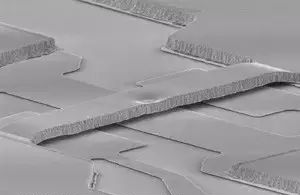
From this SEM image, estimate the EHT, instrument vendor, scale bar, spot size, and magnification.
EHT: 15 kV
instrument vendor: FEI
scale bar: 20000 nm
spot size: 3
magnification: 1.438 K X